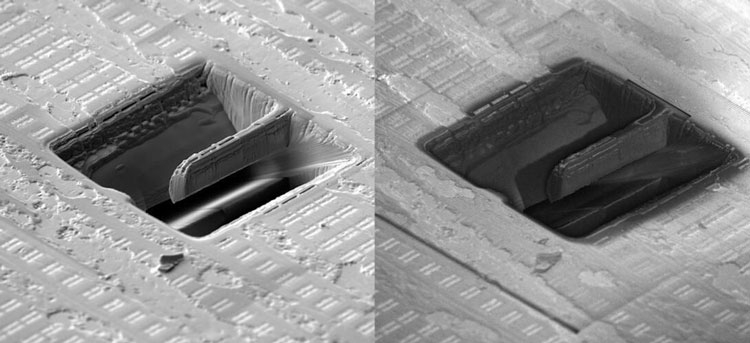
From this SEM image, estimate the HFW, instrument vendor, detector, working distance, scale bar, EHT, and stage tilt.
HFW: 140 µm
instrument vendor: Tescan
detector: E-T, Axial (BSE)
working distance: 6.05 mm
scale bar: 50000 nm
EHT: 5 kV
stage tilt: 55°